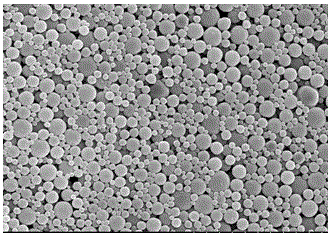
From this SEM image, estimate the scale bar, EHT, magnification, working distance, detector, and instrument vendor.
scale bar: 20000 nm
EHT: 15 kV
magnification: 2.5 K X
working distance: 7.1 mm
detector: SE(U)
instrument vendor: Hitachi